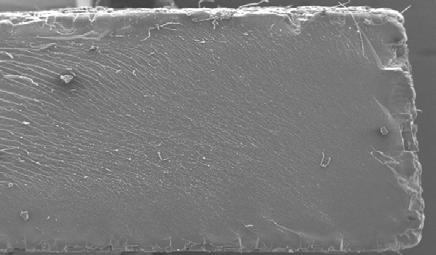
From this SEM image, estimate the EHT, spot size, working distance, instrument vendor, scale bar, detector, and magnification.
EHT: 20 kV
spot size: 5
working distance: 10.2 mm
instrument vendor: FEI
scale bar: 1000000 nm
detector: SE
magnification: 0.05 K X